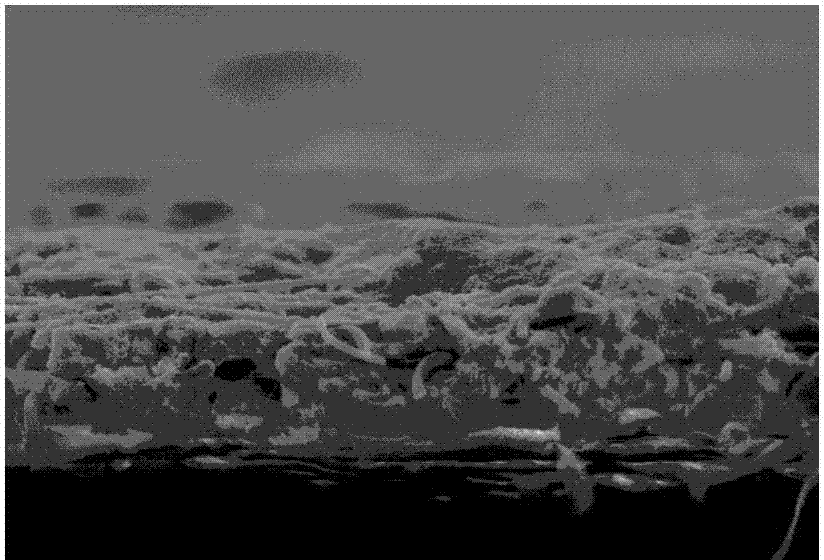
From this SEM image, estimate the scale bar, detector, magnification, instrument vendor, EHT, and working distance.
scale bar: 50000 nm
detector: SE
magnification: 0.8 K X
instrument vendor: Hitachi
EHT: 15 kV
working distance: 18.1 mm